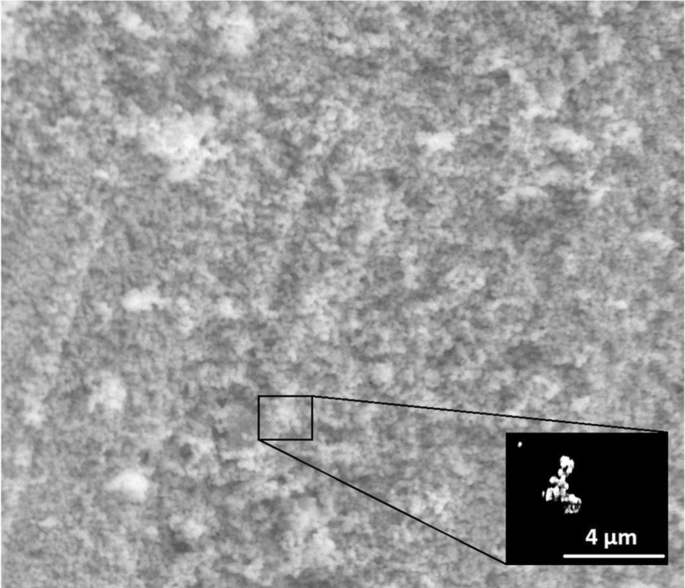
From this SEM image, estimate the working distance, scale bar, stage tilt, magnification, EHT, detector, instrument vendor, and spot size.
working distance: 9.8 mm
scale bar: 10000 nm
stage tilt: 0°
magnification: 4.452 K X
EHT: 15 kV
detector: LFD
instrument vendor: FEI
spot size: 4